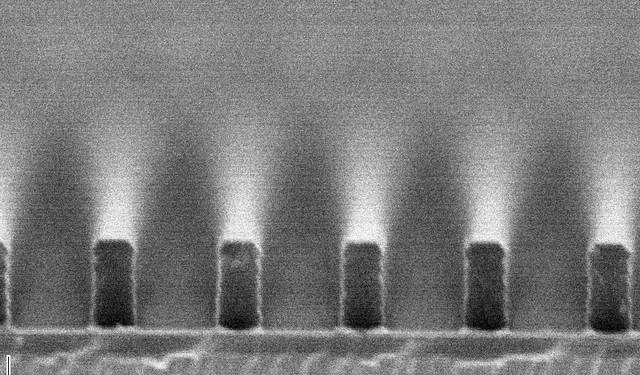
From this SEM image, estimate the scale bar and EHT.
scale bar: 1000 nm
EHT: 10 kV